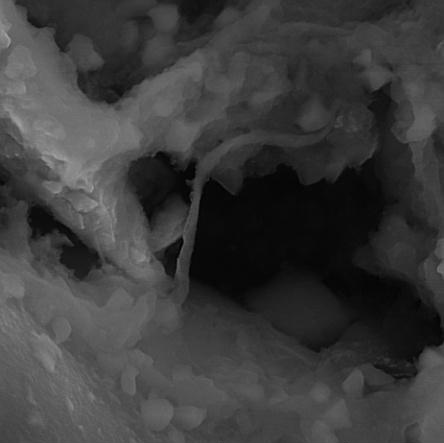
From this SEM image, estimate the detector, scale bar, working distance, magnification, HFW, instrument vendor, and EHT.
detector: InBeam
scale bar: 2000 nm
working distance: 7.63 mm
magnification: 20 K X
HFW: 7.22 µm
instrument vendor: Tescan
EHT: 15 kV